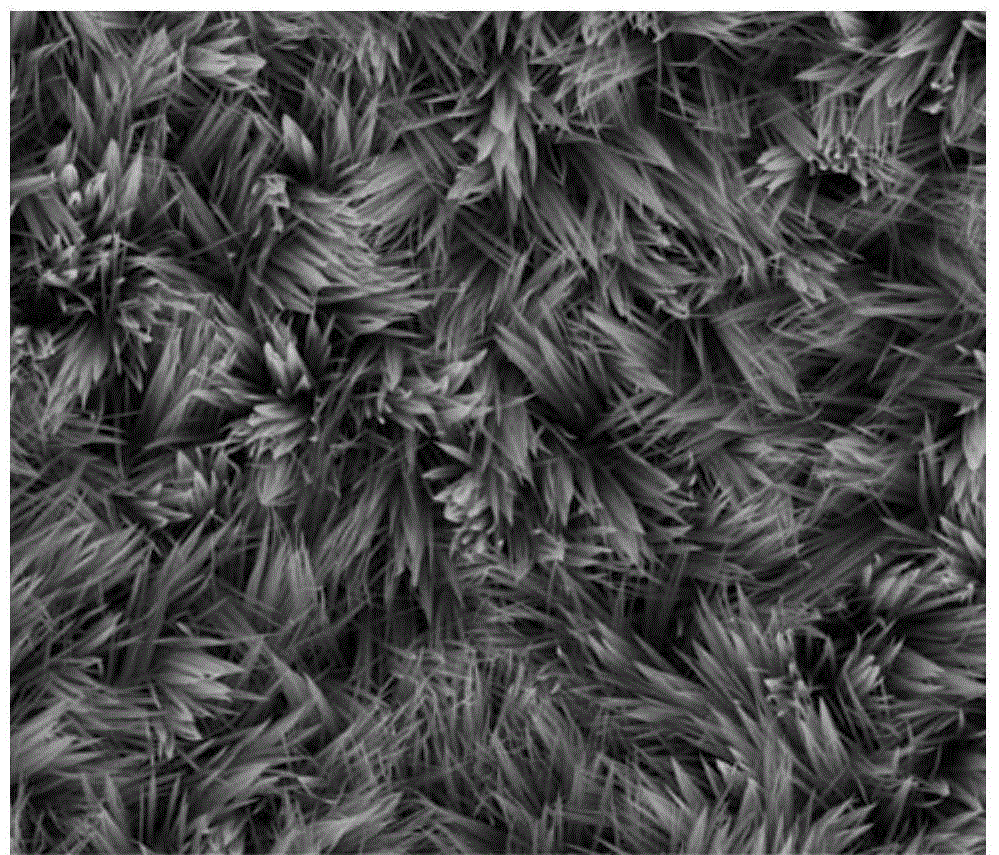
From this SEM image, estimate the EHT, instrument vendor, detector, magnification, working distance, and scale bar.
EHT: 15 kV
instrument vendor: FEI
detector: ETD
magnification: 10 K X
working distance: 4.4 mm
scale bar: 5000 nm